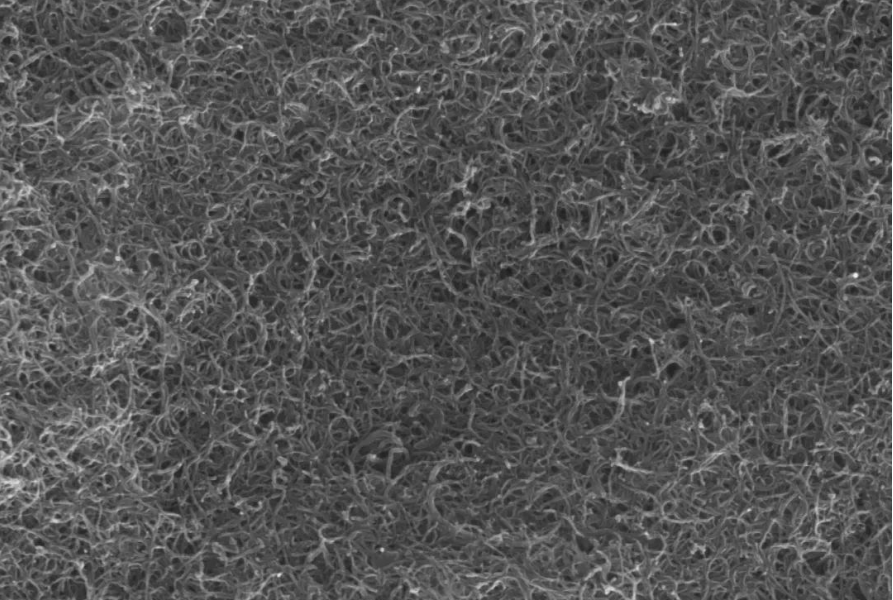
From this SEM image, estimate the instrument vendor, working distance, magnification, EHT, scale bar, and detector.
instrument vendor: other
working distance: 7.073 mm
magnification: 30 K X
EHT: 15 kV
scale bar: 1000 nm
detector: SE